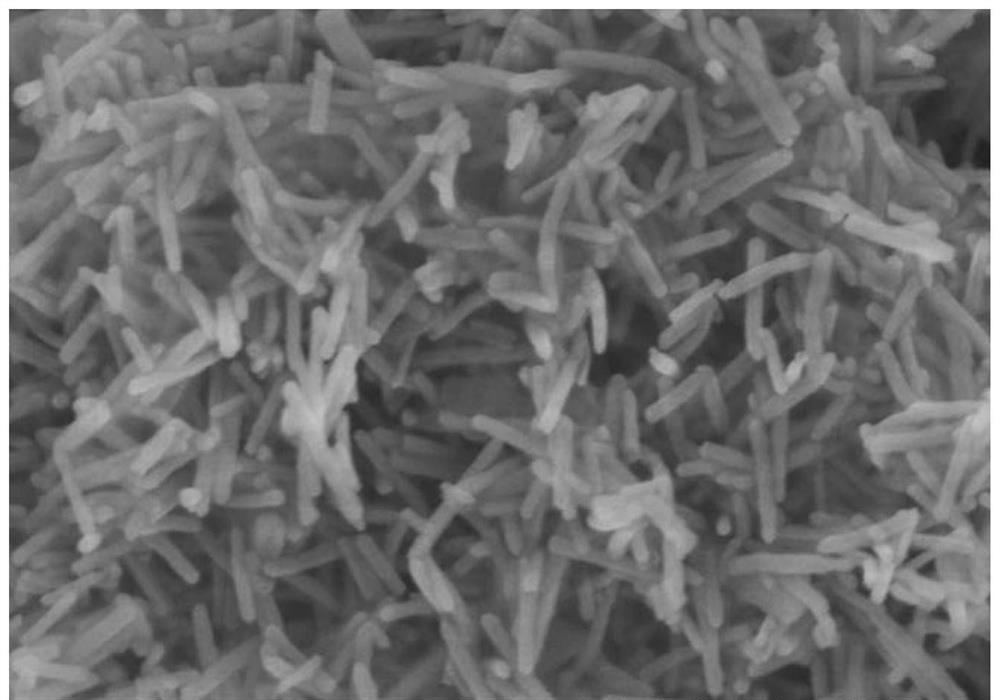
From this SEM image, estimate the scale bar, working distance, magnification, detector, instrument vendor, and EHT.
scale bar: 500 nm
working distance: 5.2 mm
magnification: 100 K X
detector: SE(U)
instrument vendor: Hitachi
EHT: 5 kV